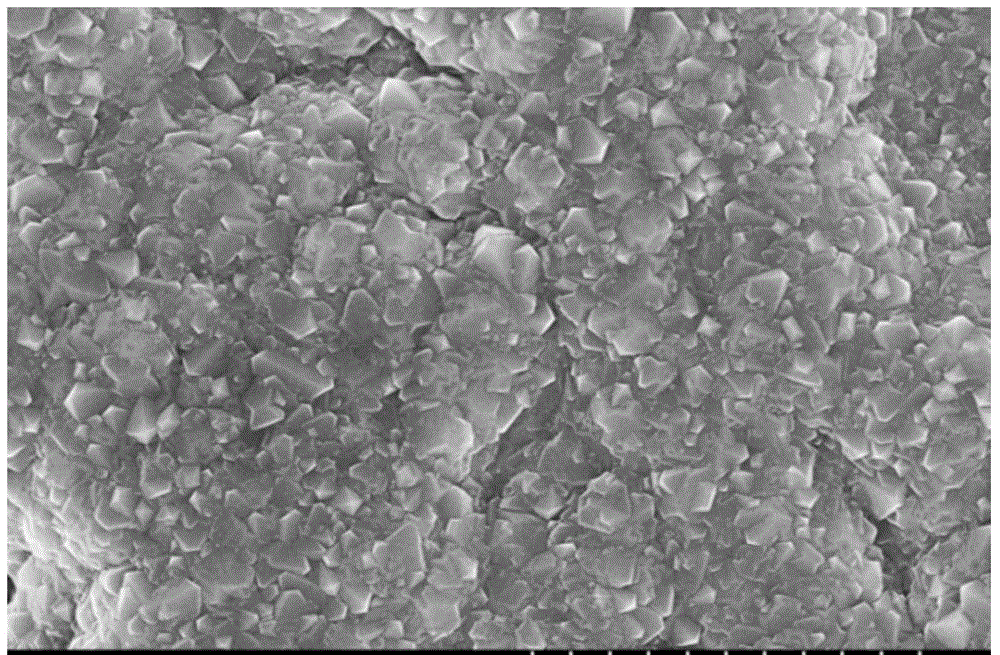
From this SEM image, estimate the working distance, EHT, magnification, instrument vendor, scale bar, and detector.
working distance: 8.9 mm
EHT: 10 kV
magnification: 10 K X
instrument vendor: Hitachi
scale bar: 5000 nm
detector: SE(M)